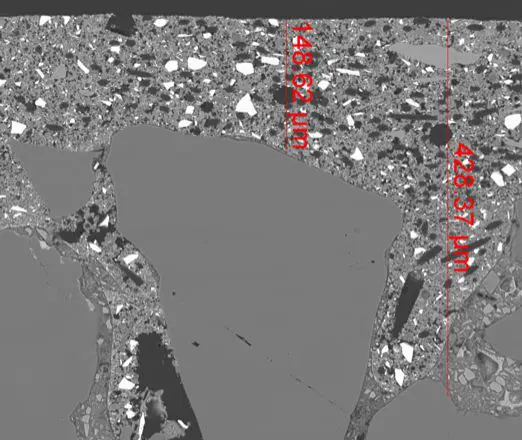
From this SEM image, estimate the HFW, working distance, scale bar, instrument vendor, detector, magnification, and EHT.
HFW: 597 µm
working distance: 9.8 mm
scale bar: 100000 nm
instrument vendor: FEI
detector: vCD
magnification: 0.5 K X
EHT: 20 kV